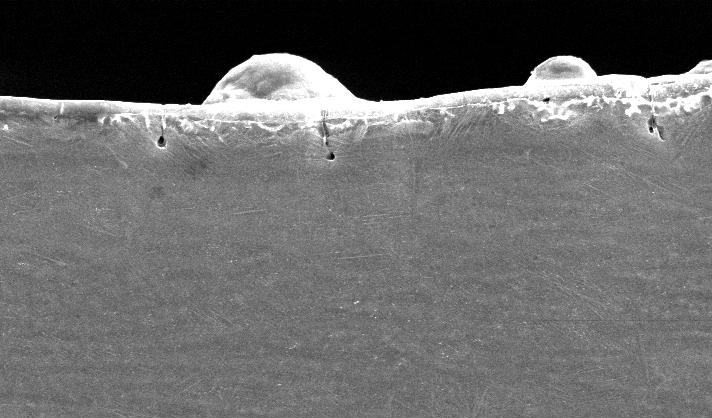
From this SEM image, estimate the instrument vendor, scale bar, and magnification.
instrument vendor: FEI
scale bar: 20000 nm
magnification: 1 K X